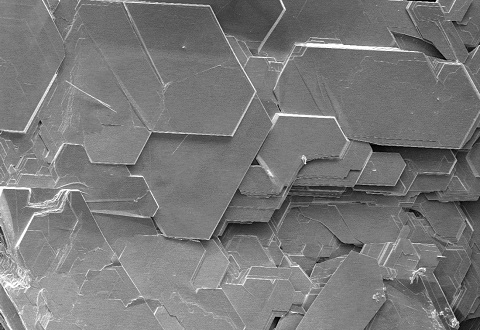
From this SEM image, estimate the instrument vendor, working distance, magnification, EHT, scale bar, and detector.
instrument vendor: Hitachi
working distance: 7.9 mm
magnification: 0.2 K X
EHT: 10 kV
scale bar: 200000 nm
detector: LM(UL)